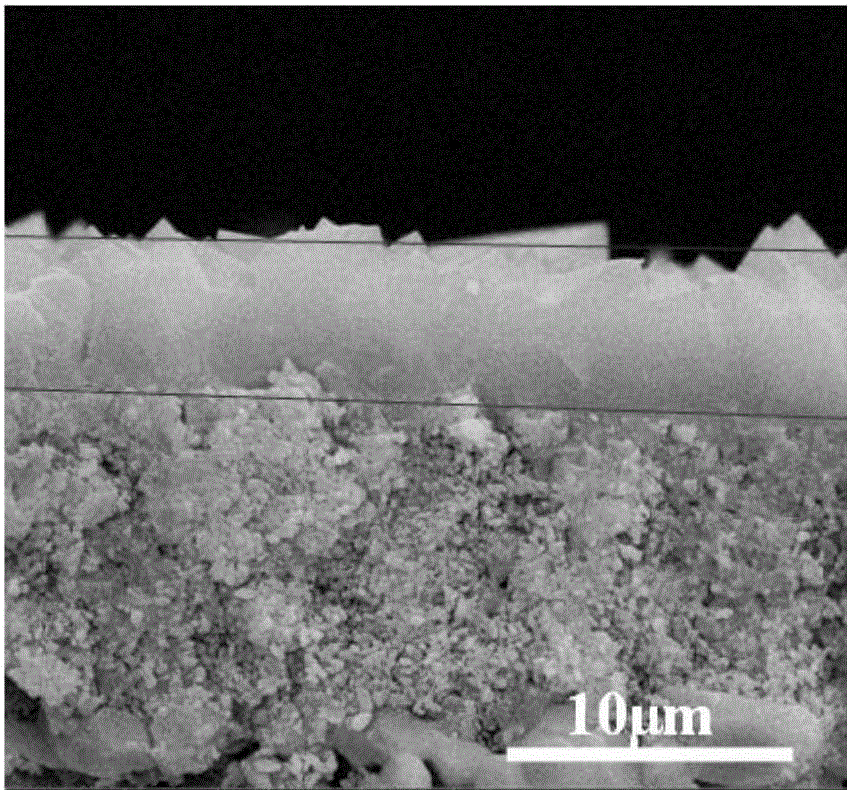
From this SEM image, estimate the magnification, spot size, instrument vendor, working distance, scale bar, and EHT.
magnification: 10 K X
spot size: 3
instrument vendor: FEI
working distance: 11.9 mm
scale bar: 10000 nm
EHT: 20 kV